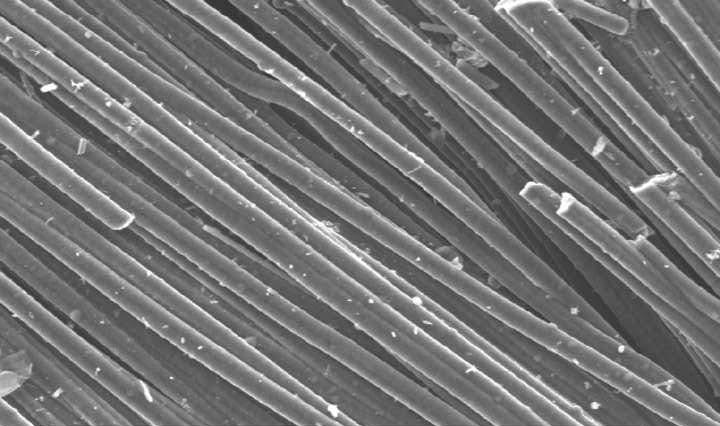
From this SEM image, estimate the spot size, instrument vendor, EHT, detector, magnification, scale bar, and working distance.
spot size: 5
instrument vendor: FEI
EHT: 20 kV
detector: SE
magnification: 0.25 K X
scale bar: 100000 nm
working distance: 10.3 mm